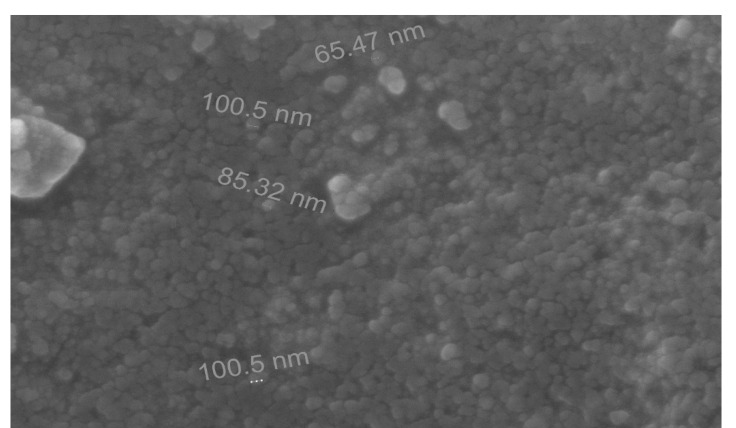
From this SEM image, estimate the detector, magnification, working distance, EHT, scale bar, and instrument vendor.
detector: ETD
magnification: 30 K X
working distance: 12.5 mm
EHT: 30 kV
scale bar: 2000 nm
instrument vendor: FEI